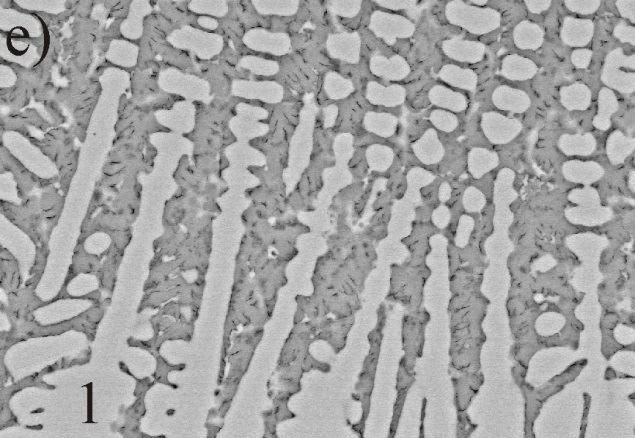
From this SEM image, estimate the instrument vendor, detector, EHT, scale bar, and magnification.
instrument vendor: Hitachi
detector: YAGBSE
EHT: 20 kV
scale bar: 10000 nm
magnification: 3 K X